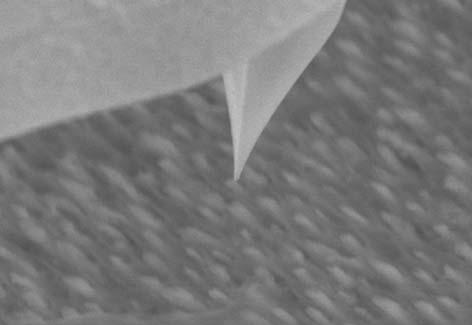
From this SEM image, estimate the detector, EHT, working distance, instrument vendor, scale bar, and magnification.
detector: SE(M)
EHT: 10 kV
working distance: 11.6 mm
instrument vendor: Hitachi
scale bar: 3000 nm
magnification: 18 K X